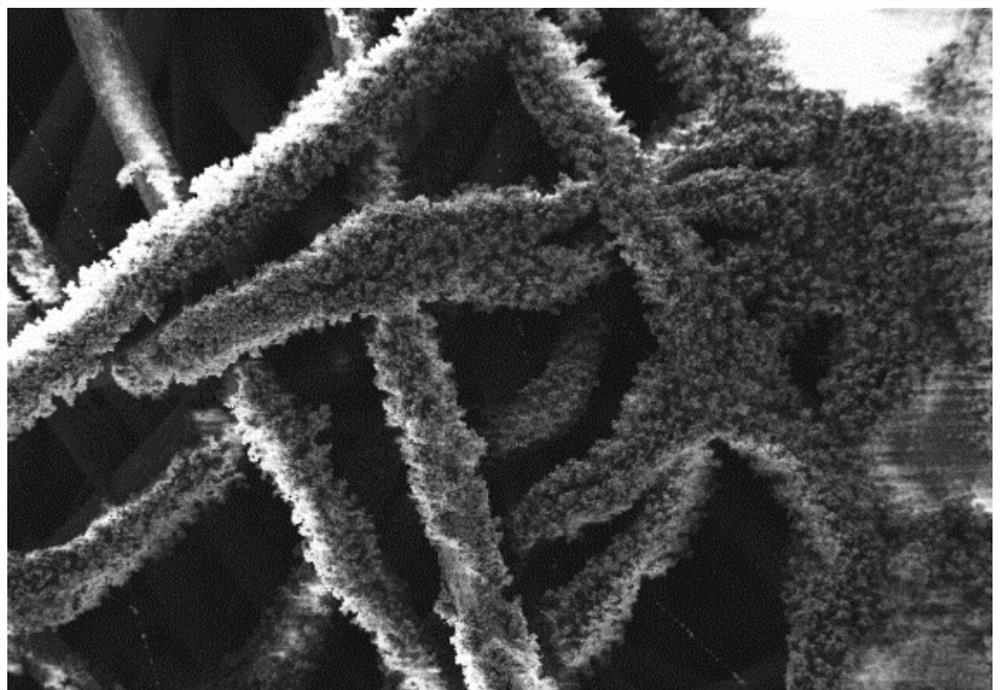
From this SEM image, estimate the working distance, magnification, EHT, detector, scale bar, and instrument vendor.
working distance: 5.7 mm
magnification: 0.266 K X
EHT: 1 kV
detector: SE2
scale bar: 100000 nm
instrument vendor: Zeiss